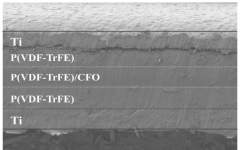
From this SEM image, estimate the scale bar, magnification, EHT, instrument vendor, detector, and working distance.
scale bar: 200000 nm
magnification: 0.22 K X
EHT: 3 kV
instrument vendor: Hitachi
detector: SE(M)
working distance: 7.8 mm